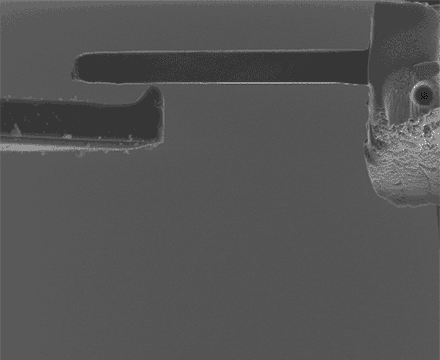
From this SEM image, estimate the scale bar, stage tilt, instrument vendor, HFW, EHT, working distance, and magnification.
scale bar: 20000 nm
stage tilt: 0°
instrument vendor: FEI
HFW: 51.2 µm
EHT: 10 kV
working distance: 5 mm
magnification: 2.5 K X